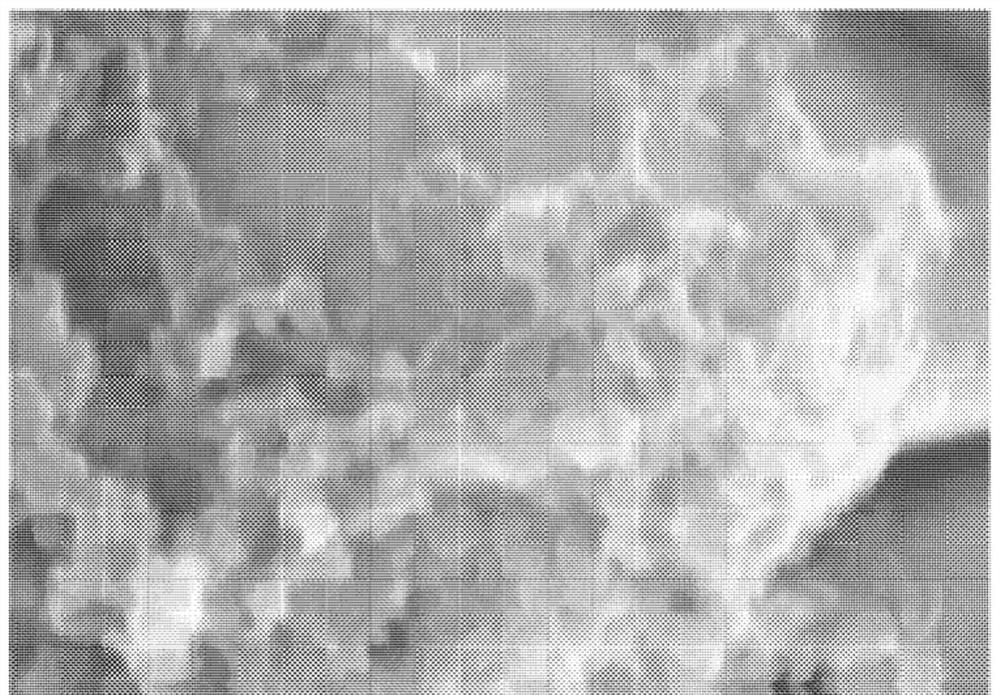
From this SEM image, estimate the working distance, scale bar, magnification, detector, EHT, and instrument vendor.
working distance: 9.4 mm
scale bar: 500 nm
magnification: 100 K X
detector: SE(M)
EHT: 5 kV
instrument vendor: Hitachi